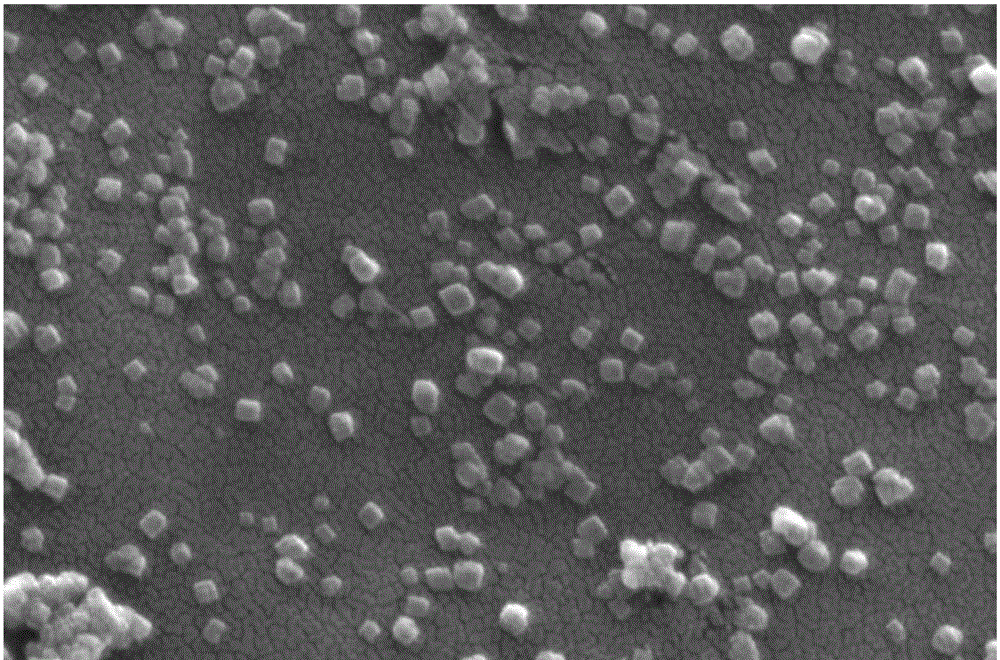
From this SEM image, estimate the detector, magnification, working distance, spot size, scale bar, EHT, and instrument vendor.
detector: SE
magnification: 100 K X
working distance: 11.4 mm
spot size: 2.5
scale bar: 500 nm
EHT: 20 kV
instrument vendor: FEI